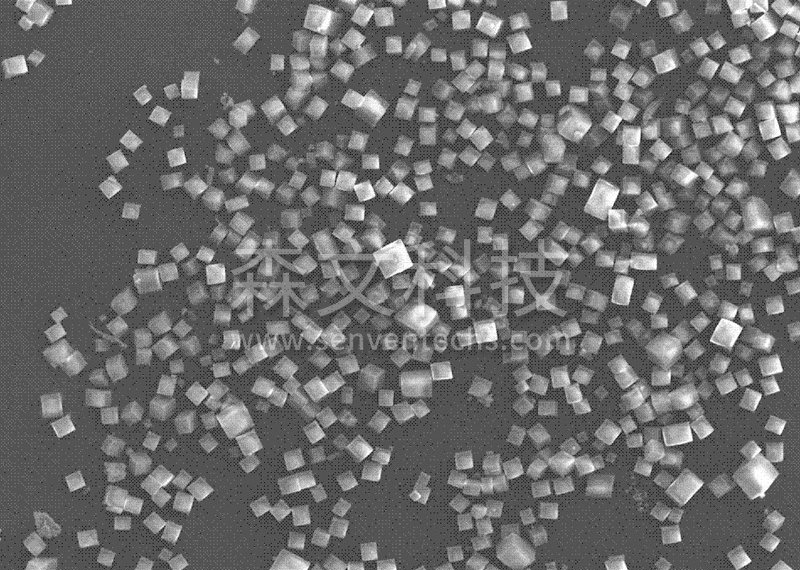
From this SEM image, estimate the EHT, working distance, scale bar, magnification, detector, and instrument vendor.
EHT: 10 kV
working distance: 17 mm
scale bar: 50000 nm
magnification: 0.5 K X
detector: SEI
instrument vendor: JEOL